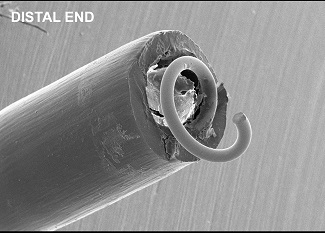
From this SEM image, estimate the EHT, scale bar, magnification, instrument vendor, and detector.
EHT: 10 kV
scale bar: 200000 nm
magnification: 0.05 K X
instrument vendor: JEOL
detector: SE